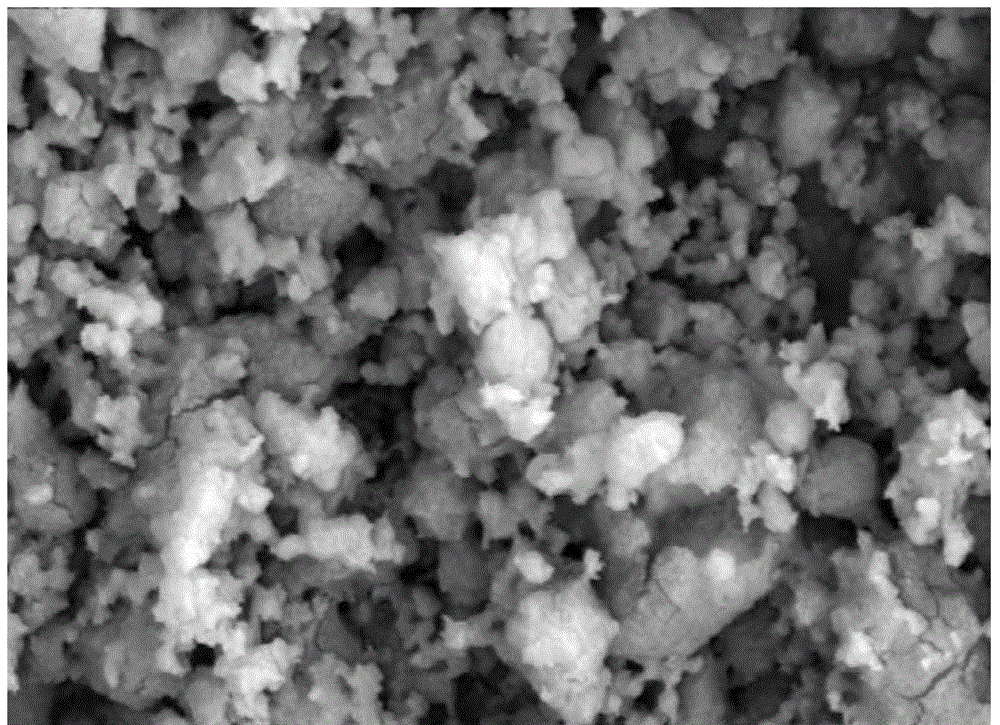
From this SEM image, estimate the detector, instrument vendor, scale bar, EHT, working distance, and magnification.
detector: SE(L)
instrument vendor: Hitachi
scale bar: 10000 nm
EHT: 15 kV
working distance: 12.4 mm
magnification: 5 K X